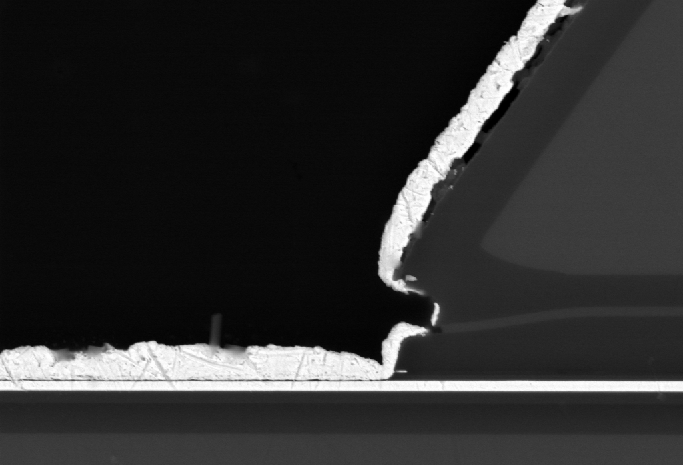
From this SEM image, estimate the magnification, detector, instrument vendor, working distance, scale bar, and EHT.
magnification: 10 K X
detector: CZ BSD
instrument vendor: Zeiss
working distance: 6.8 mm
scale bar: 2000 nm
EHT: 10 kV